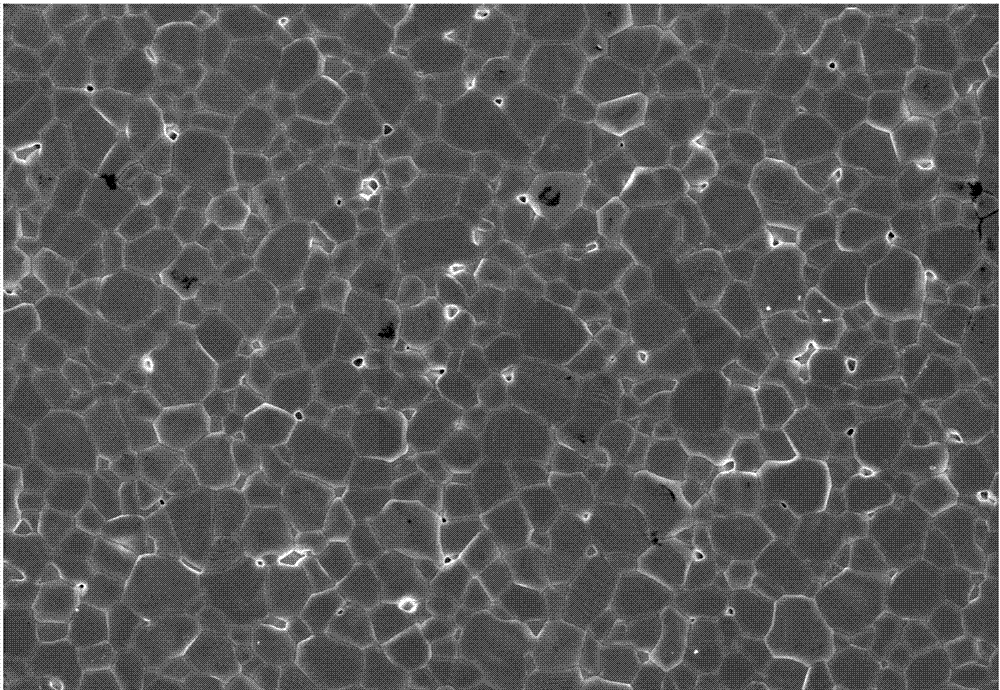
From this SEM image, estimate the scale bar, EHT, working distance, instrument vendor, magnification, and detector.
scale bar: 50000 nm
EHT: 10 kV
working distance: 8.5 mm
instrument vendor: Hitachi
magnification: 1 K X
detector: SE(U)